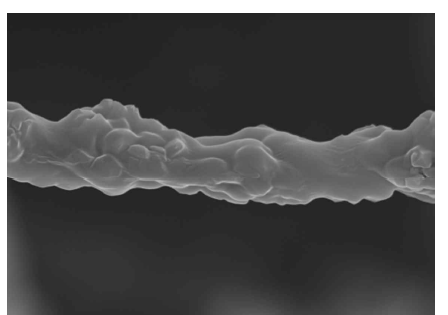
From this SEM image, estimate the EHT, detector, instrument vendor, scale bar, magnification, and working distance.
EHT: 10 kV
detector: SEI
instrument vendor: JEOL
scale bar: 1000 nm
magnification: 10 K X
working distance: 7.8 mm